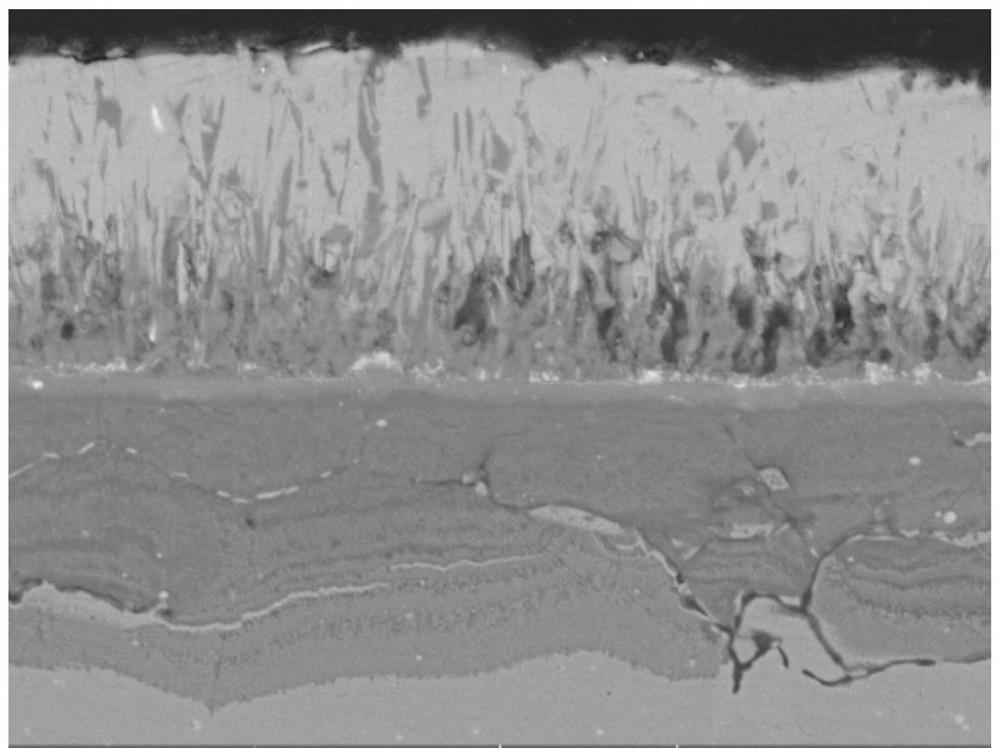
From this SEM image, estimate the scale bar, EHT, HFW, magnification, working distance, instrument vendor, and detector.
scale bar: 10000 nm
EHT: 15 kV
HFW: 55.4 µm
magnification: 5 K X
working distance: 14.64 mm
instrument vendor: Tescan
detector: BSE